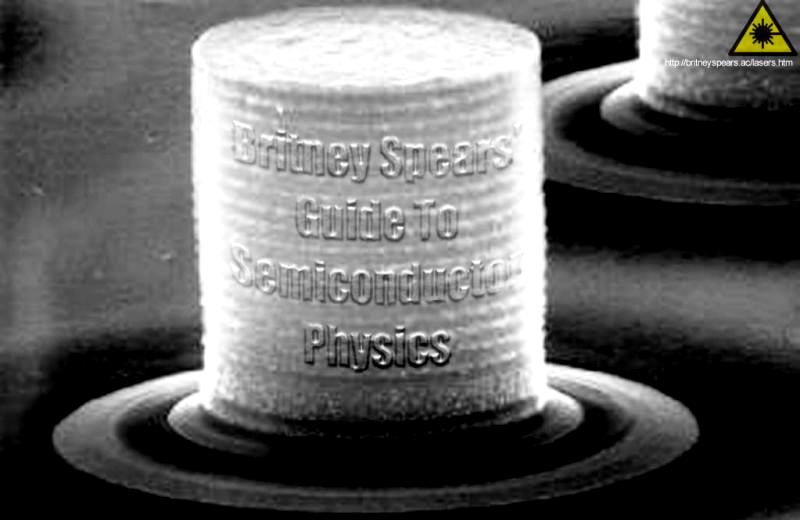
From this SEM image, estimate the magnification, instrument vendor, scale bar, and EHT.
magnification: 18 K X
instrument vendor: JEOL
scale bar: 1670 nm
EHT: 25 kV